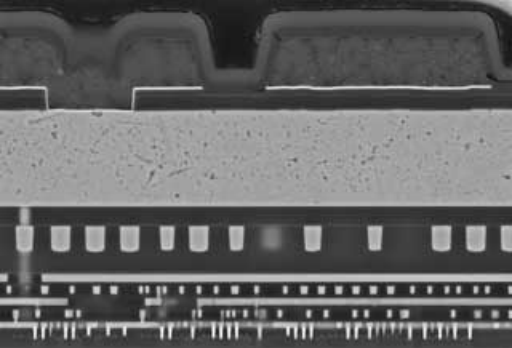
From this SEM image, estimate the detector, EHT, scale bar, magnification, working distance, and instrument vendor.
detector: LA50(U)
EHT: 10 kV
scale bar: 5000 nm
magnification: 7 K X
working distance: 4.4 mm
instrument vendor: Hitachi